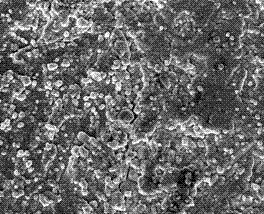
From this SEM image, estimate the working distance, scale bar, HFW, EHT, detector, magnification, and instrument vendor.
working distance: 6.9 mm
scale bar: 10000 nm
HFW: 49.7 µm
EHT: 15 kV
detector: ETD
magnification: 3 K X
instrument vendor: FEI